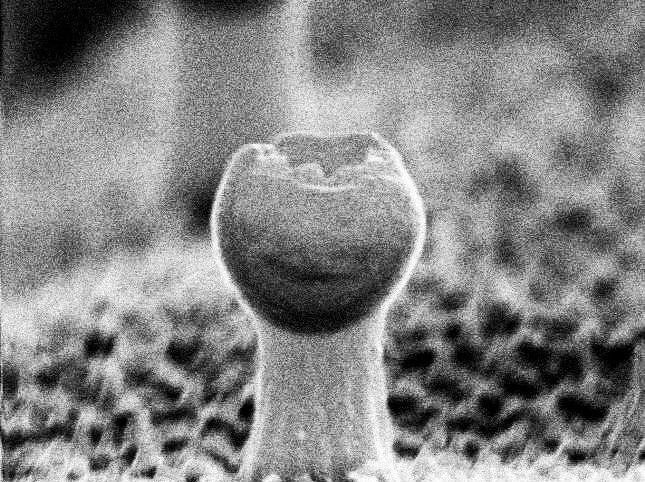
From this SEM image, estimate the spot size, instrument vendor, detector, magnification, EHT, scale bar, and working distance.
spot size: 3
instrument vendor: FEI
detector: TLD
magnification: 127.2 K X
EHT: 30 kV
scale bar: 200 nm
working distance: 6.2 mm